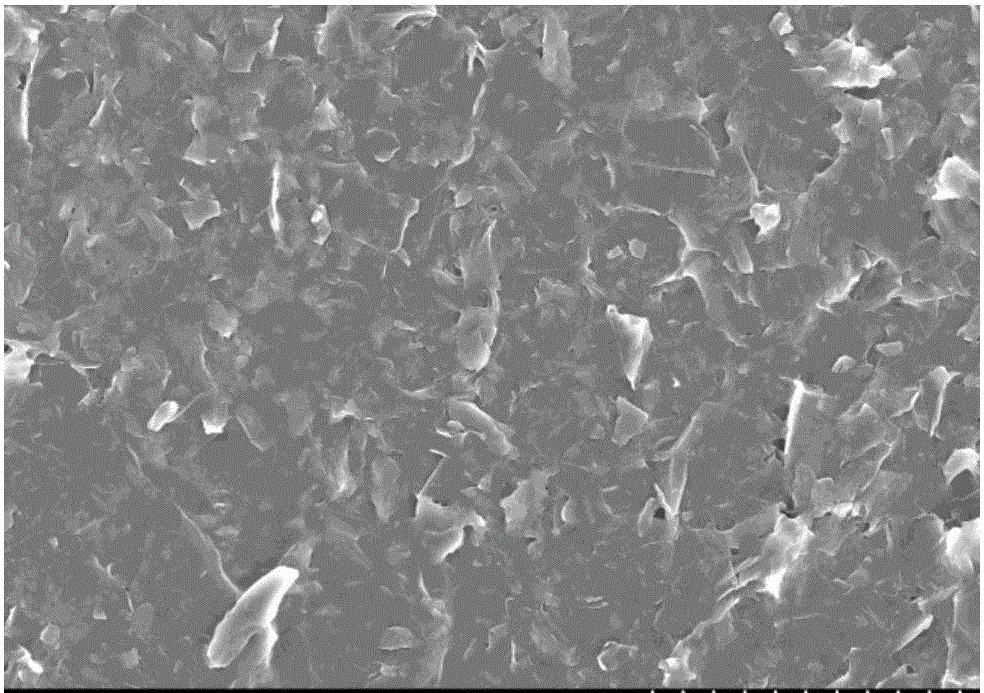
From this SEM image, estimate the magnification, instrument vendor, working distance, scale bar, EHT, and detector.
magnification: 2 K X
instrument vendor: Hitachi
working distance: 8.3 mm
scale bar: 20000 nm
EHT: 10 kV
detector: SE(M)